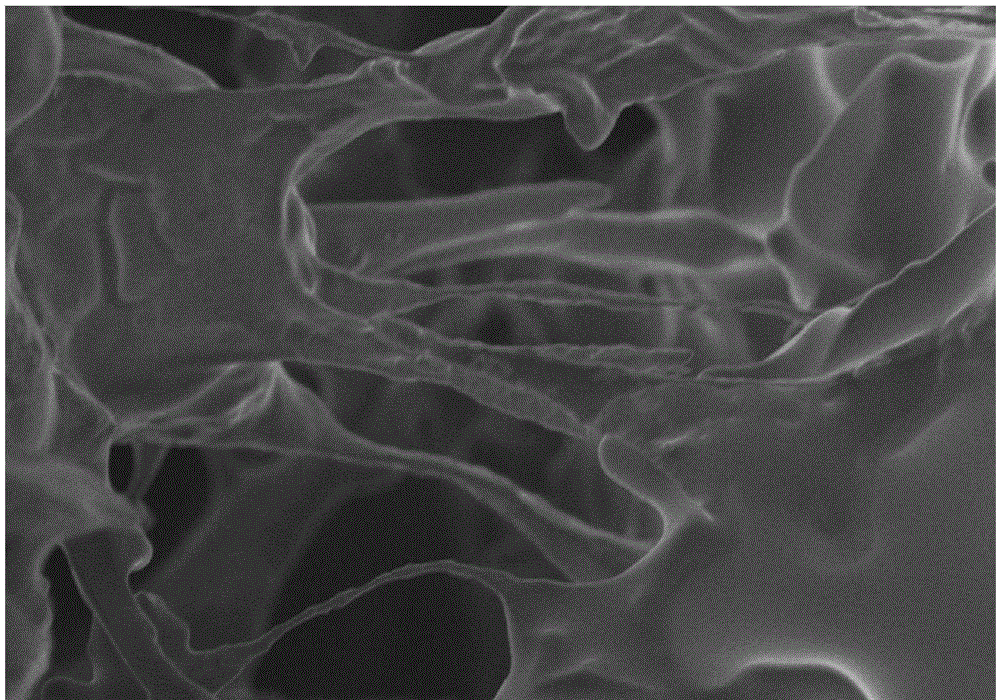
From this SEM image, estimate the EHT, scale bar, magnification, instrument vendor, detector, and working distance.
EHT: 2 kV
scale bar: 20000 nm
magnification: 2 K X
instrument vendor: Hitachi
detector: SE(M)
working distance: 12.8 mm